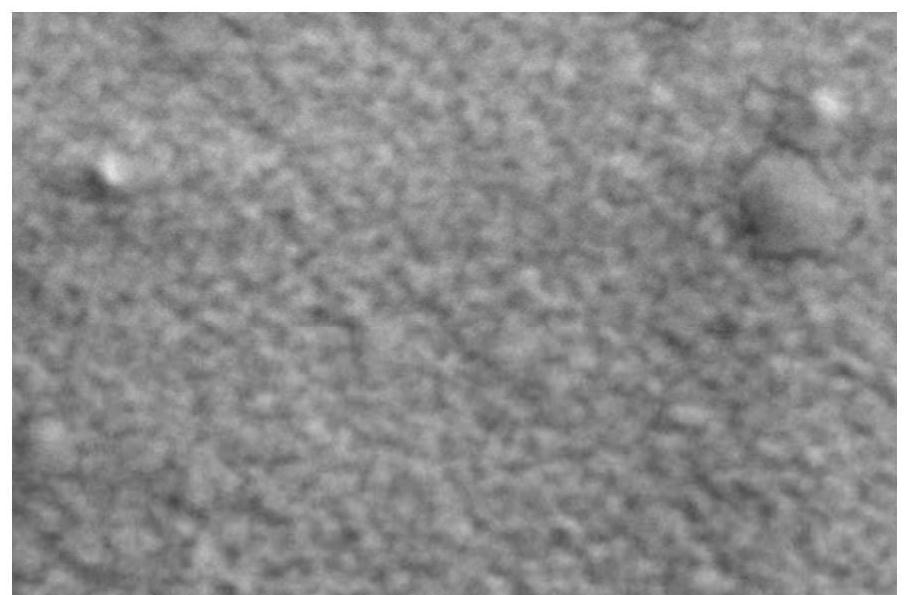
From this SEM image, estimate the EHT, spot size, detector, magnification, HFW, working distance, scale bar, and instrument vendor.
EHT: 2 kV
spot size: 10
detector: ETD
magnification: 100 K X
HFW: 1.27 µm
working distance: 10.9 mm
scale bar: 300 nm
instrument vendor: Thermo